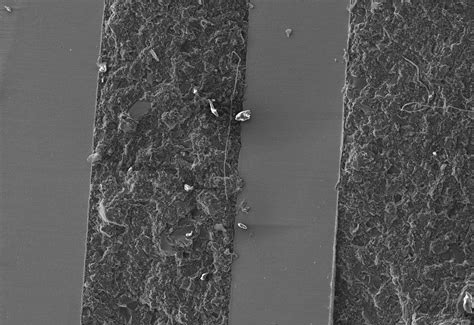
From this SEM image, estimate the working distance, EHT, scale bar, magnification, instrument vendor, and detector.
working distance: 12.3 mm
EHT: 15 kV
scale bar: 500000 nm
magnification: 0.1 K X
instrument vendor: Hitachi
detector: SE(L)